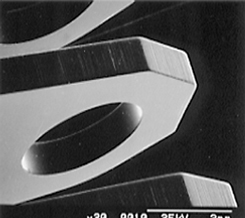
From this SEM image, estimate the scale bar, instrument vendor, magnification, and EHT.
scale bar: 2000000 nm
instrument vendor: JEOL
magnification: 0.02 K X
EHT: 25 kV